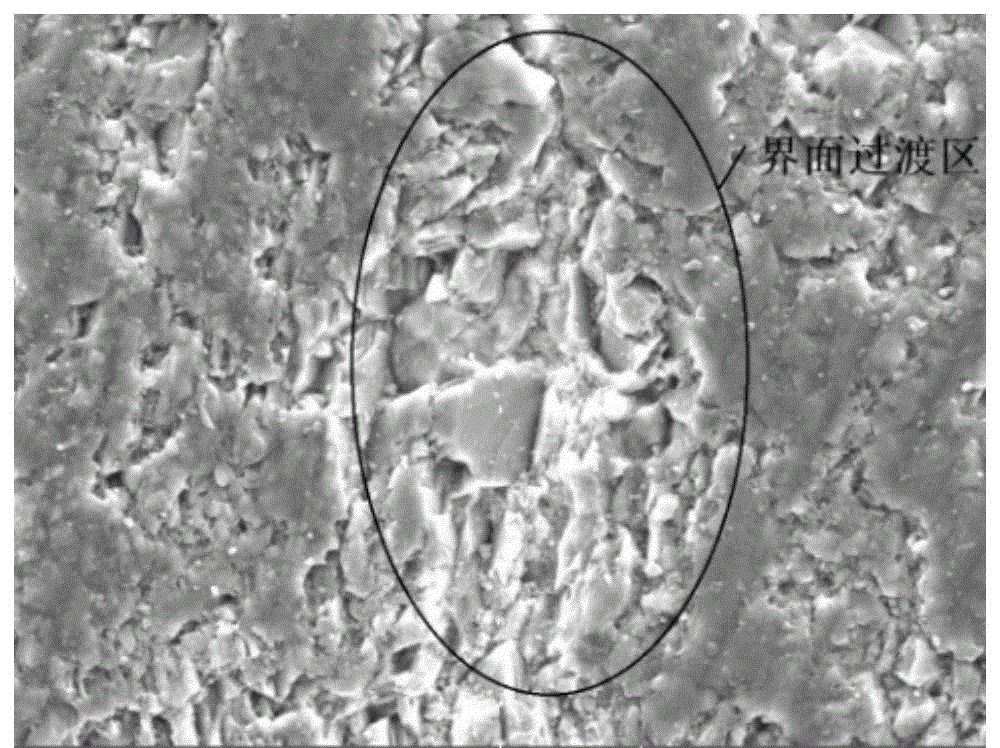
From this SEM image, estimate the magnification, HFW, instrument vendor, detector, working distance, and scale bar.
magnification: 2 K X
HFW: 138 µm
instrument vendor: Tescan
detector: SE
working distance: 31.08 mm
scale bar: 20000 nm